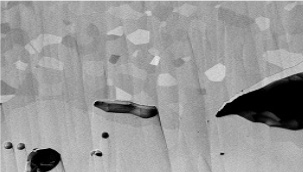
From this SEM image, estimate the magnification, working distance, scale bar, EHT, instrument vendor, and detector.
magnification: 5 K X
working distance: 7.8 mm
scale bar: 10000 nm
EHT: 5 kV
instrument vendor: Hitachi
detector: PDBSE